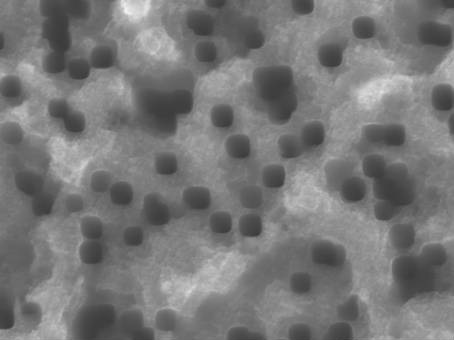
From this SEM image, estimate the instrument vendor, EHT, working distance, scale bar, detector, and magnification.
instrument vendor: JEOL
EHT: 15 kV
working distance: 6.7 mm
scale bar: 100 nm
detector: SEI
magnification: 30 K X